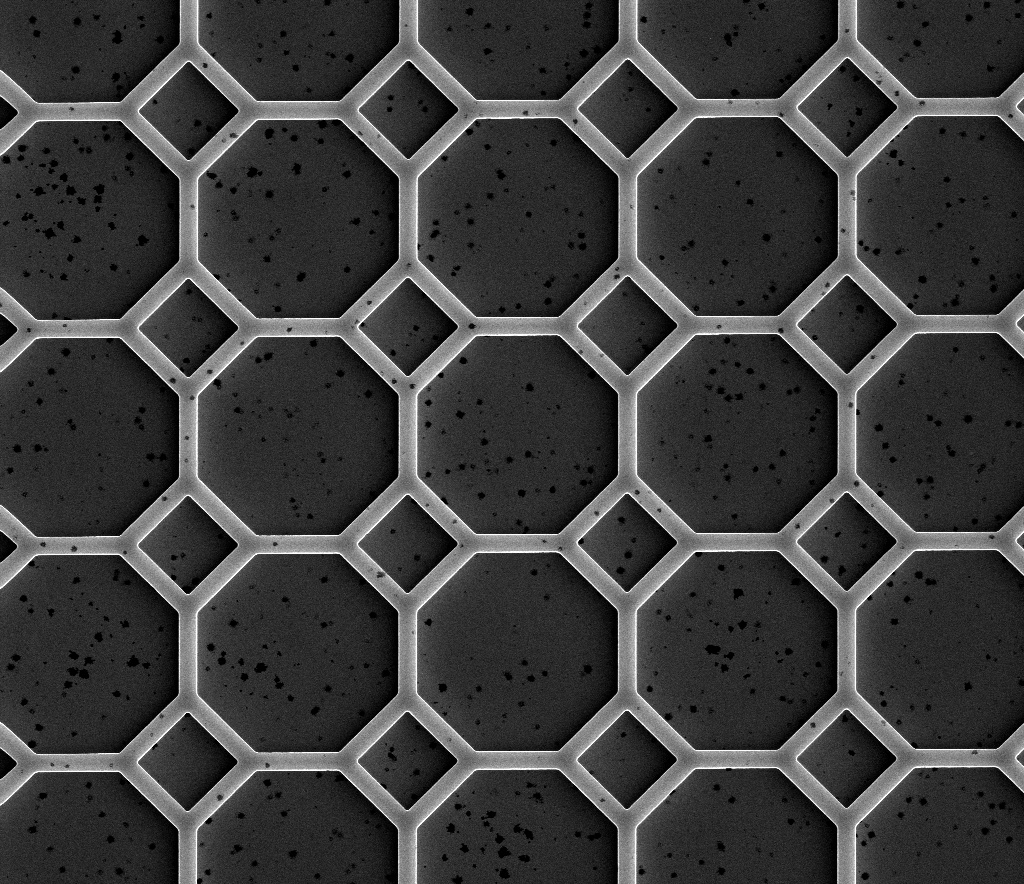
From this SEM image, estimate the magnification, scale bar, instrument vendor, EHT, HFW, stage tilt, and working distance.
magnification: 0.5 K X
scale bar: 50000 nm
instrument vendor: FEI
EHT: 3.2 kV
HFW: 256 µm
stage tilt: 0°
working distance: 9.3 mm